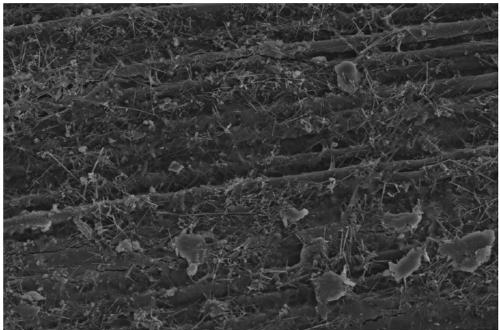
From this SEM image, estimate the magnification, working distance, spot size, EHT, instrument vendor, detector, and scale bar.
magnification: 0.8 K X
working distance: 5.5 mm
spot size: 3.5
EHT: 15 kV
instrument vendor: FEI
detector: ETD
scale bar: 50000 nm